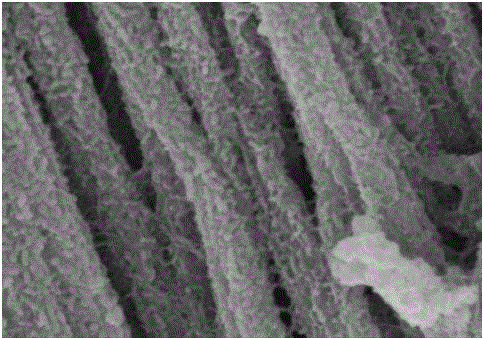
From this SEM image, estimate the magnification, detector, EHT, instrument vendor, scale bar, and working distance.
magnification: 110 K X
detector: SE(M)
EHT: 5 kV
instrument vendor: Hitachi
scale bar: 500 nm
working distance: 5.4 mm